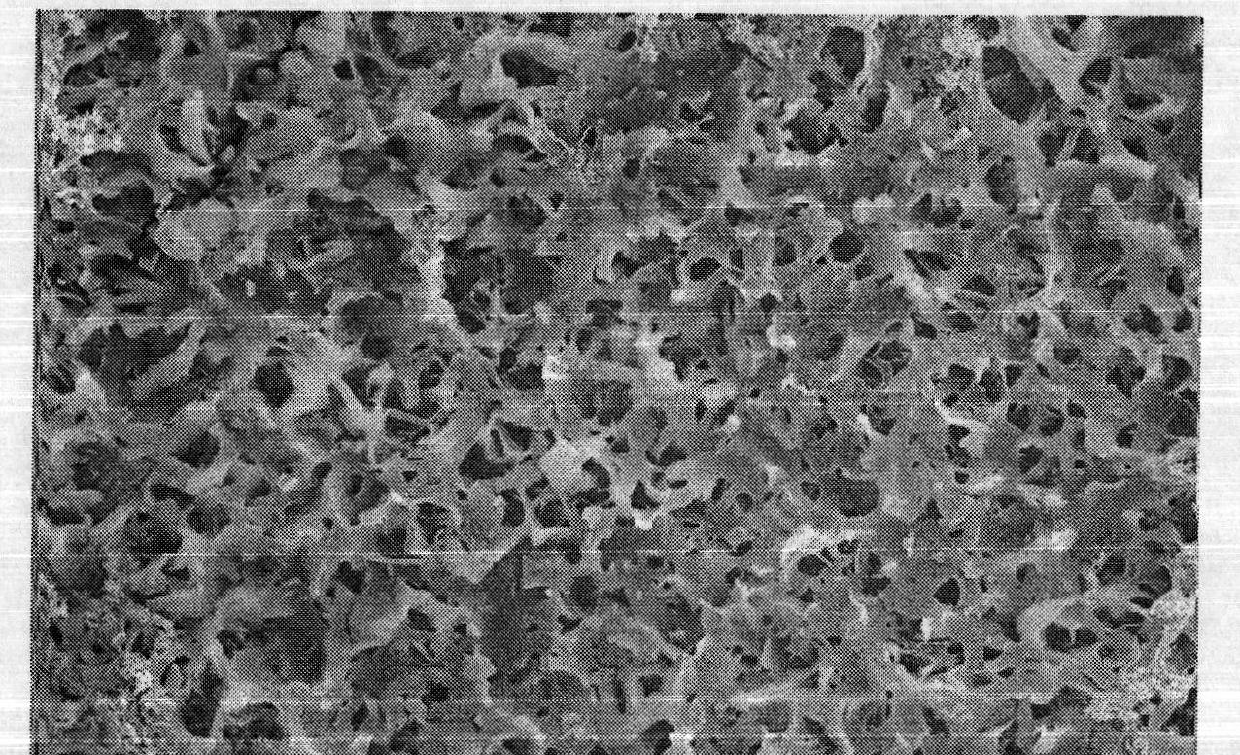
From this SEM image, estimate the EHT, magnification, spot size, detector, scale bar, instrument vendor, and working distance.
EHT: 20 kV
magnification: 10 K X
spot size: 3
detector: SE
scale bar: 5000 nm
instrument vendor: FEI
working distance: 9.1 mm